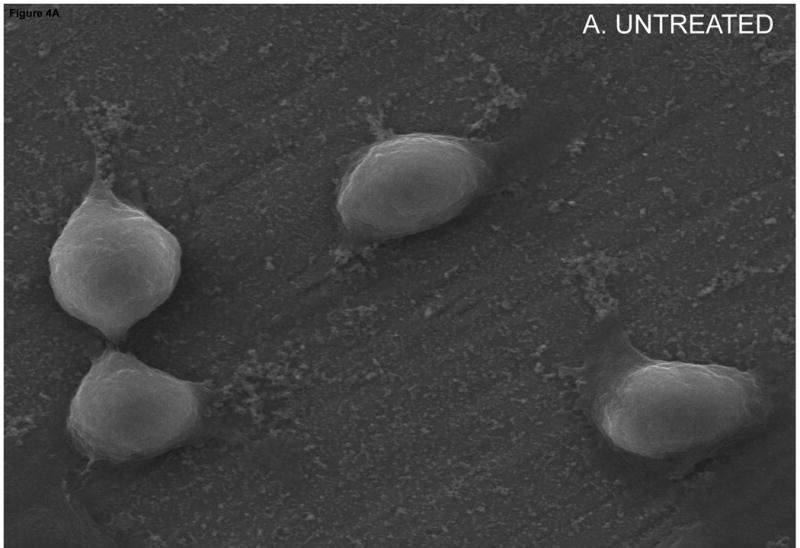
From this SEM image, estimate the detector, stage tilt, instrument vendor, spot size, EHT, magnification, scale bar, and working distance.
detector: Etd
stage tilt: -0°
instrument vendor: FEI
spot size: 3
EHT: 20 kV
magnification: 5 K X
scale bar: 10000 nm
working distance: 10.04 mm